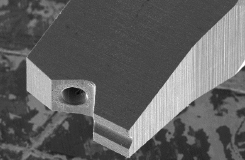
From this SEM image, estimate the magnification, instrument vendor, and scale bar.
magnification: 0.15 K X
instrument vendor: JEOL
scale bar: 100000 nm